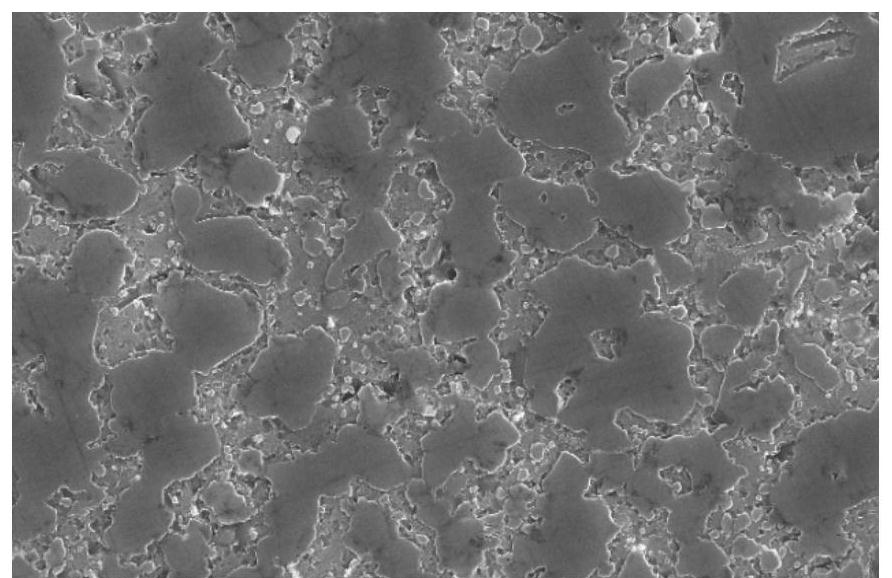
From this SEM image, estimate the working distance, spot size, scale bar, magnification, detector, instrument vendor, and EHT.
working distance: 5.9 mm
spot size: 4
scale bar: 10000 nm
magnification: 2 K X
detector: SE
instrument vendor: FEI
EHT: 20 kV